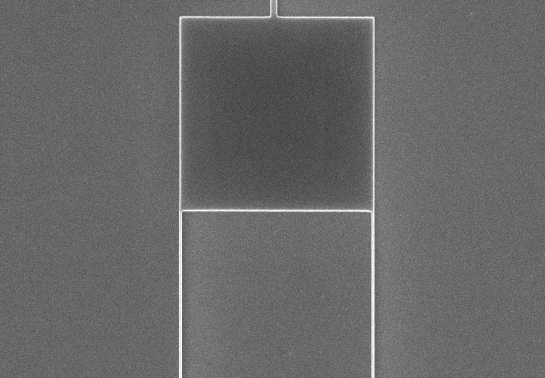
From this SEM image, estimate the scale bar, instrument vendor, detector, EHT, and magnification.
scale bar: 3000 nm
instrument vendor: Hitachi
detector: LA0(U)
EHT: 10 kV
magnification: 15 K X